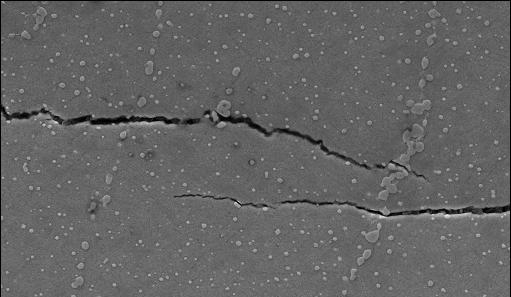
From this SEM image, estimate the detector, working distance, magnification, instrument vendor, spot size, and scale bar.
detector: SE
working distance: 11.5 mm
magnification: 1 K X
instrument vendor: FEI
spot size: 3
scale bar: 20000 nm